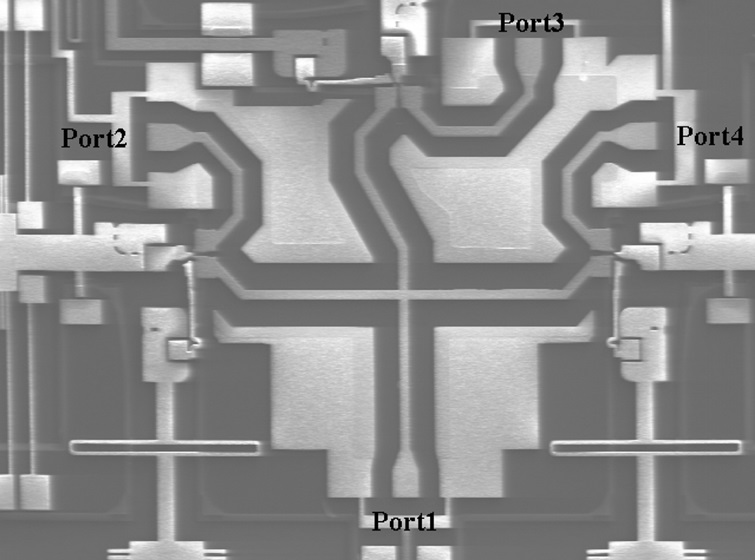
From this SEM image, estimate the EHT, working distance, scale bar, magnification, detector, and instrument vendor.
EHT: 5 kV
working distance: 23 mm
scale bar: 500000 nm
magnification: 0.06 K X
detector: SE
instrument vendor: JEOL